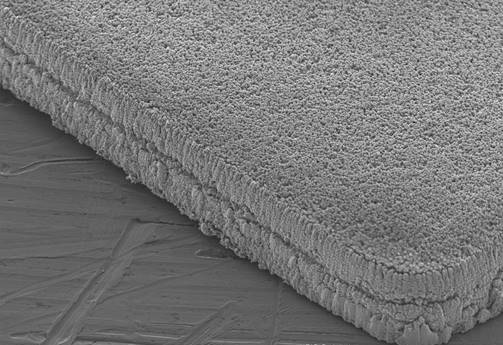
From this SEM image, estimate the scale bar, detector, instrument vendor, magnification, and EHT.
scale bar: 200000 nm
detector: SEI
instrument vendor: JEOL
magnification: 0.1 K X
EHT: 15 kV